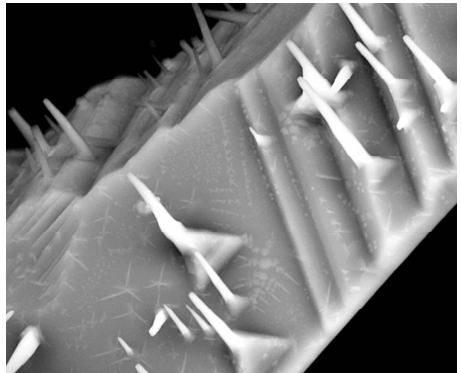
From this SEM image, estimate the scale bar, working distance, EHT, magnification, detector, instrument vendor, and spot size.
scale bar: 5000 nm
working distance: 5.6 mm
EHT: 20 kV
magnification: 10 K X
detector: BSED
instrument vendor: FEI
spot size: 5